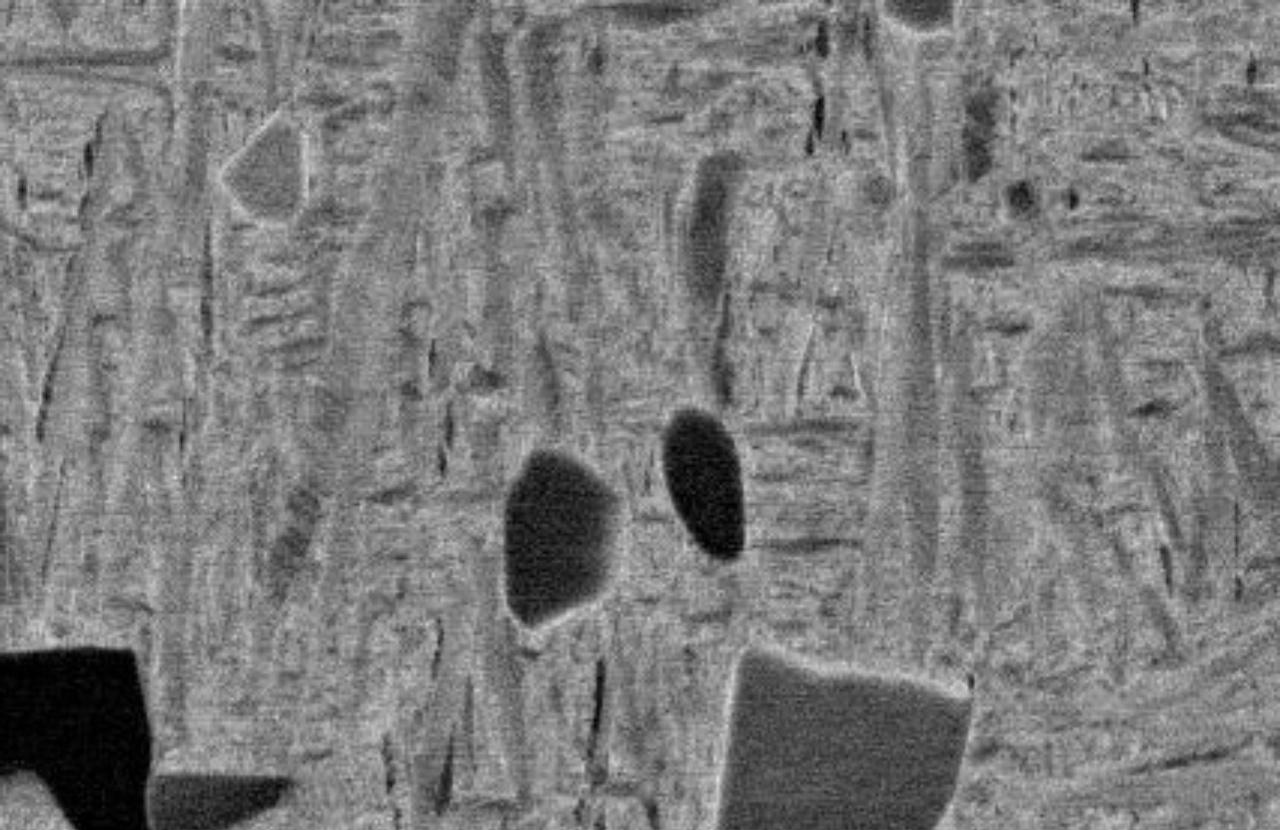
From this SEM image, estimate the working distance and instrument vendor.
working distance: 10 mm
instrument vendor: JEOL